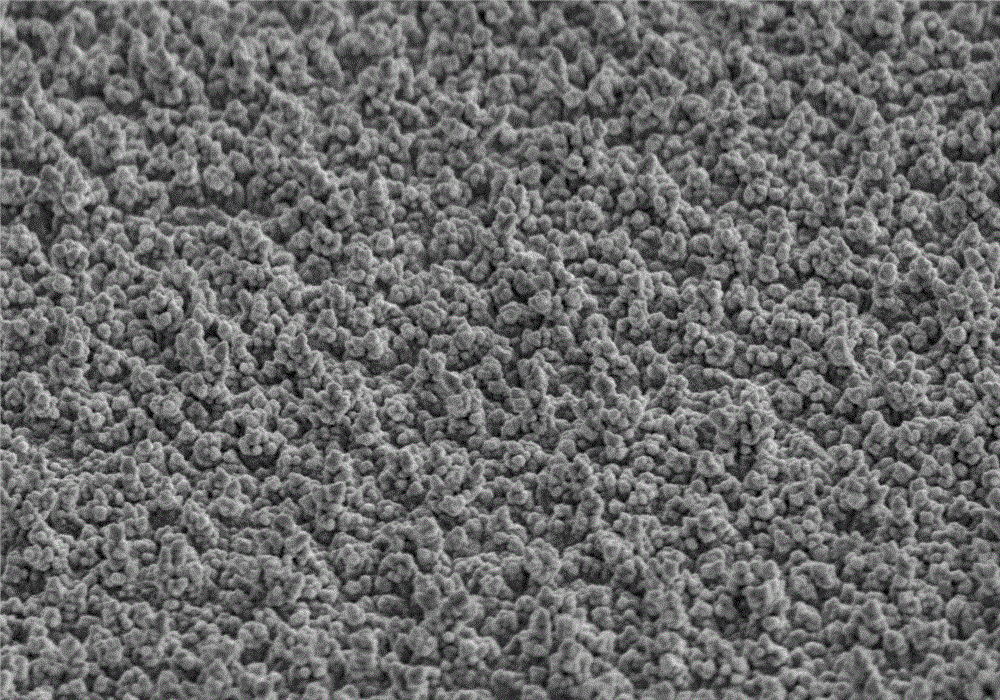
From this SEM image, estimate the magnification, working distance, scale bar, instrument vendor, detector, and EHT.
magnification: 1 K X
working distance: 19.7 mm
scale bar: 50000 nm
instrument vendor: Hitachi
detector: SE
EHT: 20 kV